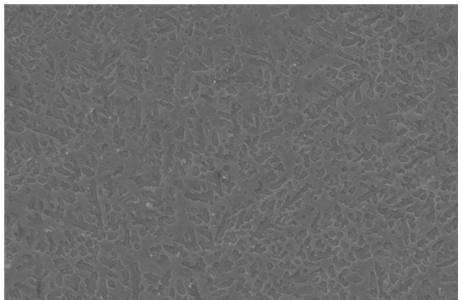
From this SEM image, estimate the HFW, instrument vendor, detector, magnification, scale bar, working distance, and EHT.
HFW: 82.9 µm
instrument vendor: FEI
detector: ETD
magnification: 5 K X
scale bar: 30000 nm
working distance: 11 mm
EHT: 25 kV